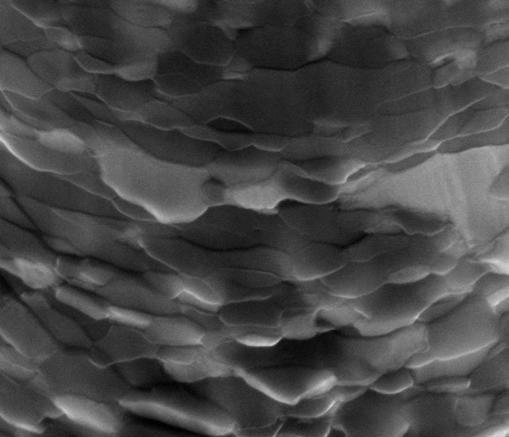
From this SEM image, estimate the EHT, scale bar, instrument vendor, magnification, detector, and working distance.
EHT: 15 kV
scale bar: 500 nm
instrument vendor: FEI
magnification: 100 K X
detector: ETD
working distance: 10 mm